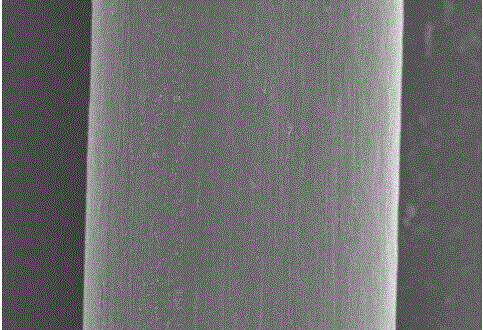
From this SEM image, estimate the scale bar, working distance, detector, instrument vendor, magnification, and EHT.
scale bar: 50000 nm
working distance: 7.6 mm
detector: SE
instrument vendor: Hitachi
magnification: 1 K X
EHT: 5 kV